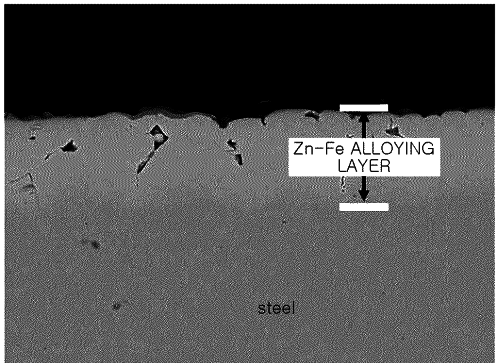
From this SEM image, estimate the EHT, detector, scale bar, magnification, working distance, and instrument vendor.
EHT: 20 kV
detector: COMPO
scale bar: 10000 nm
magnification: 1 K X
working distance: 11 mm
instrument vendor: JEOL